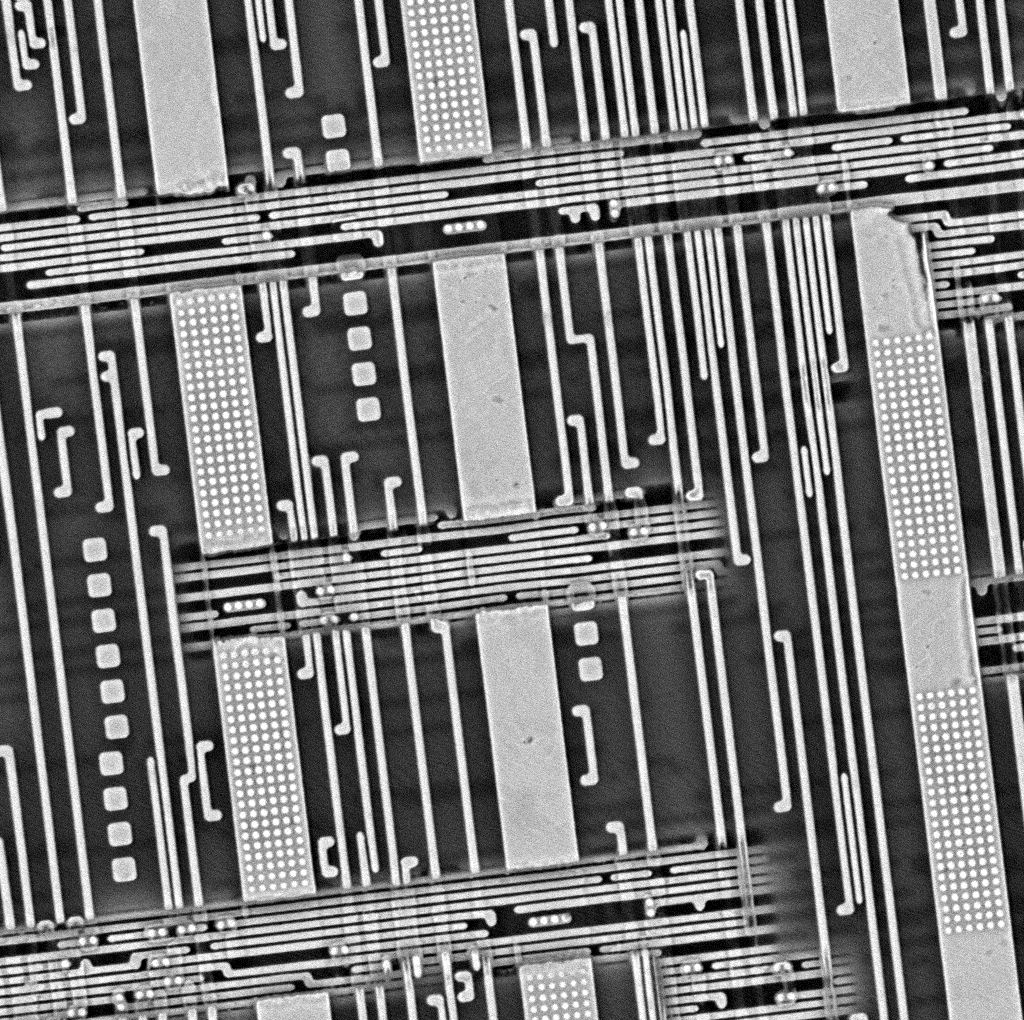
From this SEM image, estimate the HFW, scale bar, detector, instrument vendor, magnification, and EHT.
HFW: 27.1 µm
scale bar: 8000 nm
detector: BSD Full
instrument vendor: other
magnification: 10 K X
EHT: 10 kV